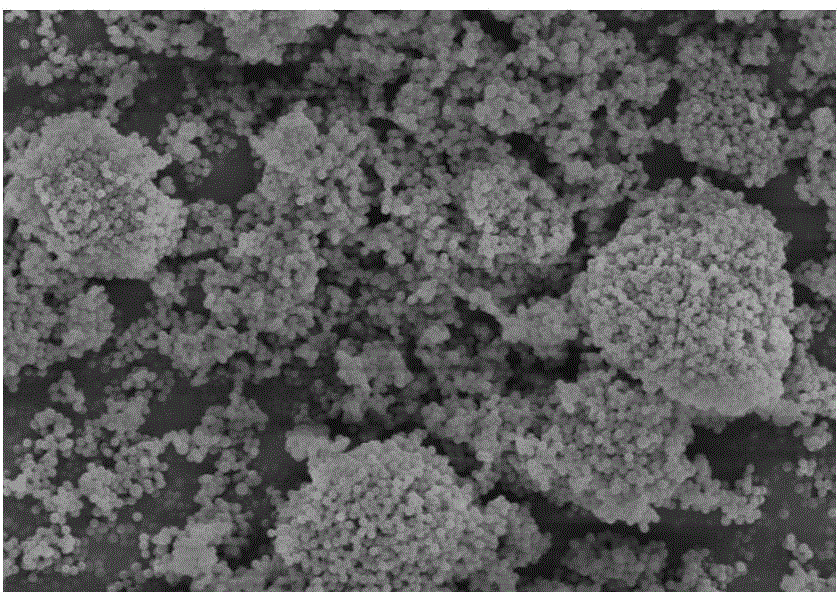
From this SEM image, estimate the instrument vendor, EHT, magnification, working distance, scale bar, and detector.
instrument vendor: Hitachi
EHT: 7 kV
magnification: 5 K X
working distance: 9.1 mm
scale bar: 10000 nm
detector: SE(M)